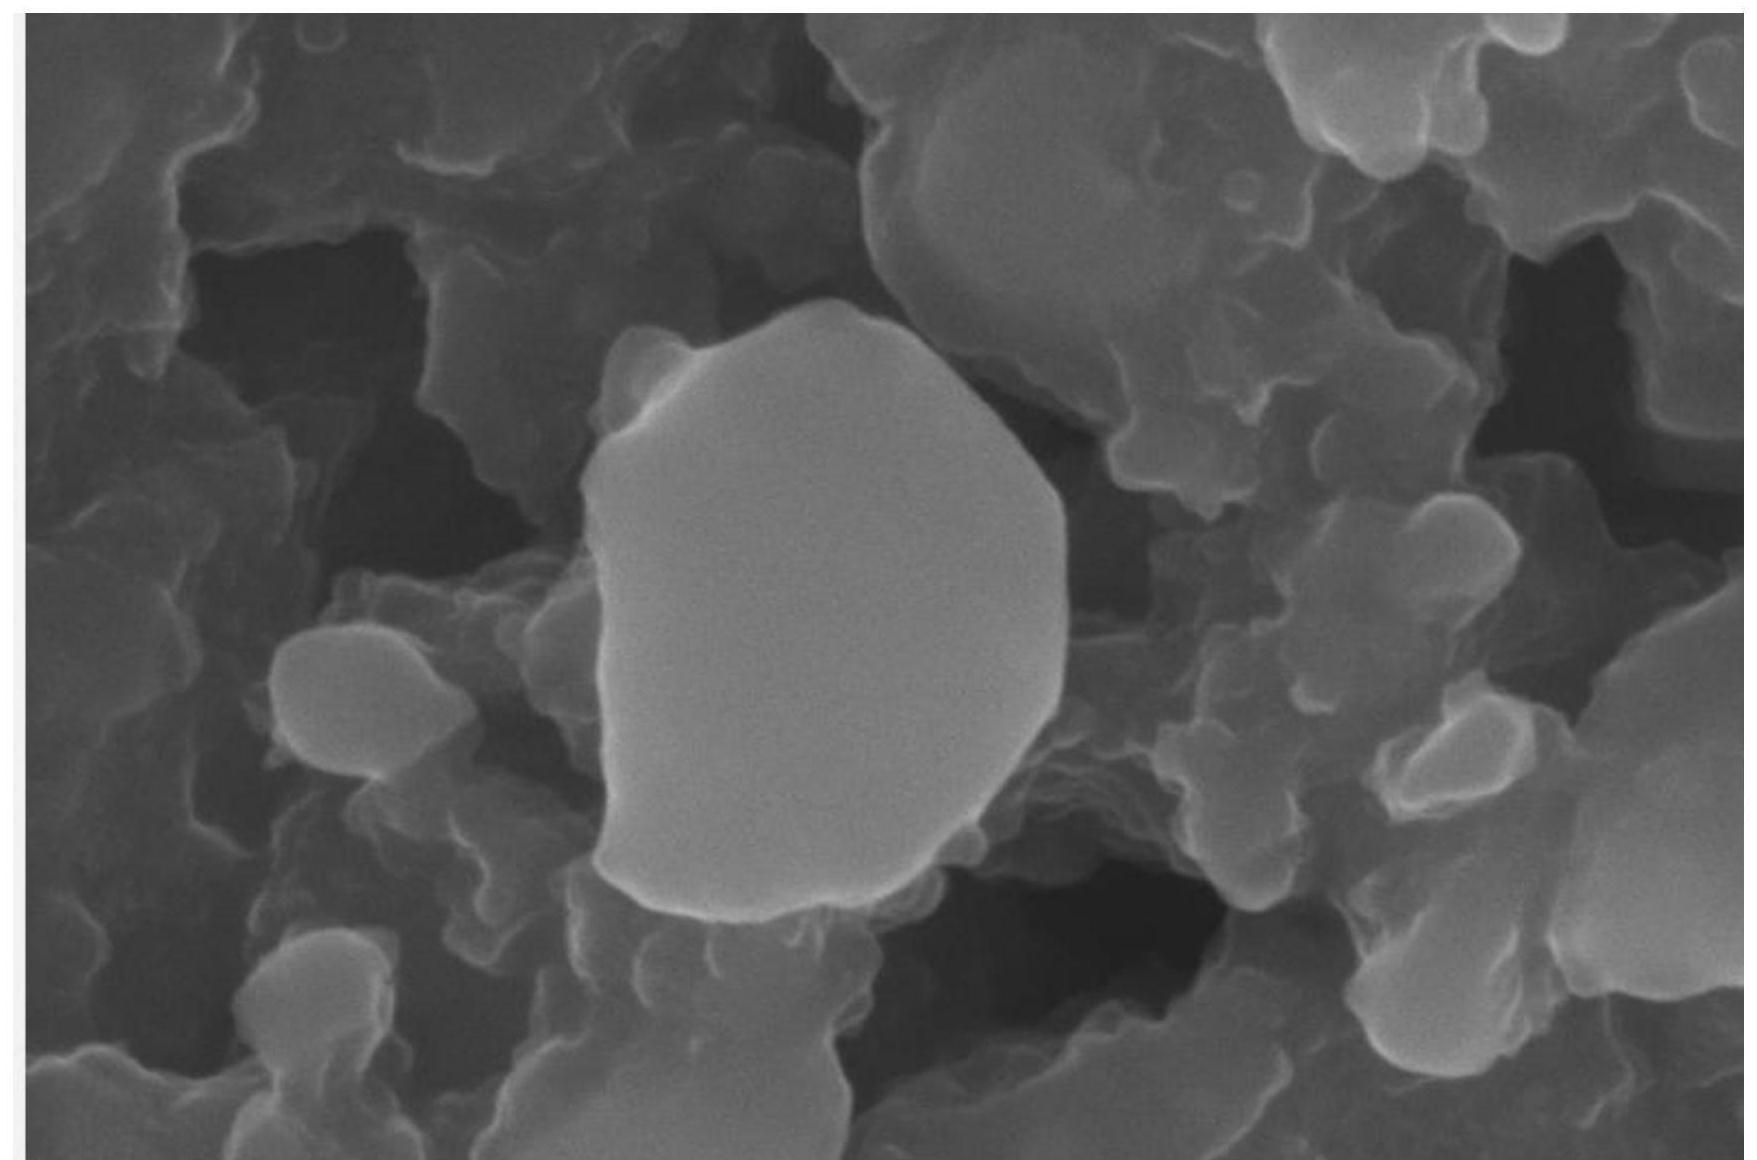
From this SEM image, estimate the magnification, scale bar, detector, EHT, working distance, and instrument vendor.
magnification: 100 K X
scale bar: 100 nm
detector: InLens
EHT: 10 kV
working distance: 7 mm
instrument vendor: Zeiss